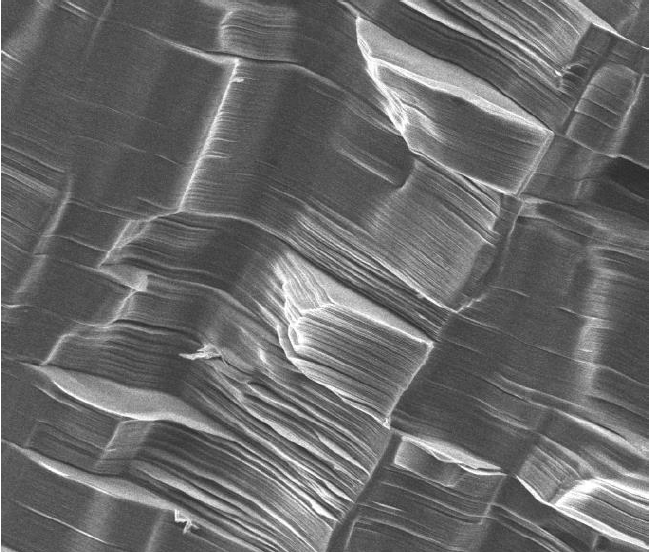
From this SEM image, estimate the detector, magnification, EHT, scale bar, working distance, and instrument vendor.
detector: ETD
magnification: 1 K X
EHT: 15 kV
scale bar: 50000 nm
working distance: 5.7 mm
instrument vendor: FEI